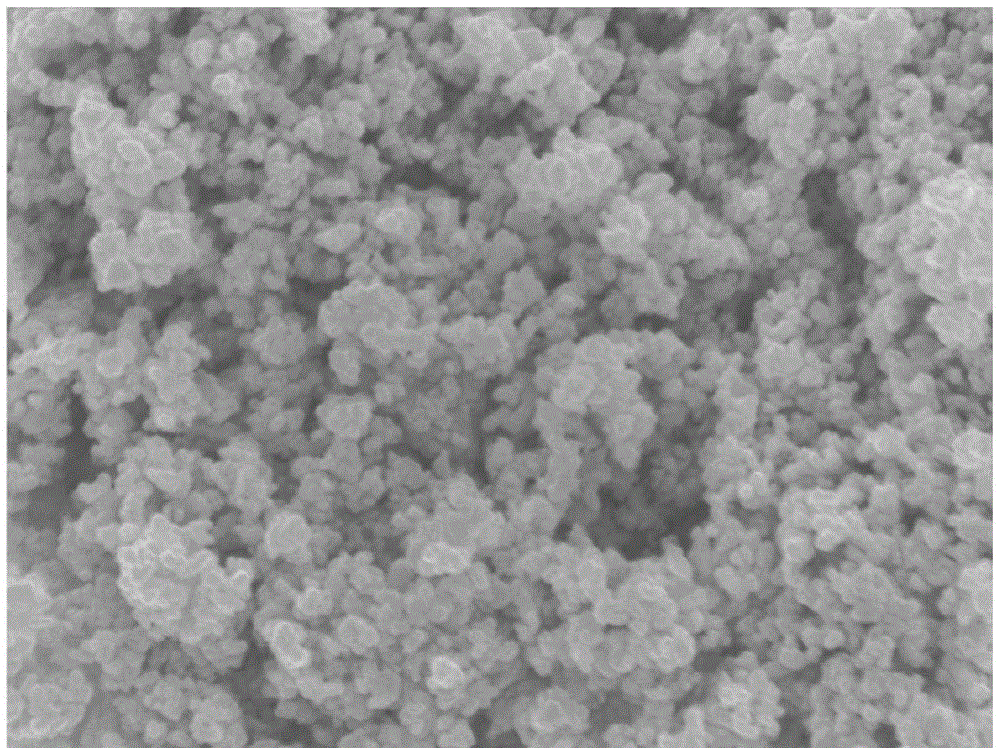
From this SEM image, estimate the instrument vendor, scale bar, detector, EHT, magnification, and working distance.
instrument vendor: JEOL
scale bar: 100 nm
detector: SEI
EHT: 5 kV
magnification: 35 K X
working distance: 7.9 mm